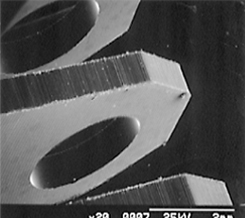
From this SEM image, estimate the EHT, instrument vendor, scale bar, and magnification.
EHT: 25 kV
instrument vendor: JEOL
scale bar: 2000000 nm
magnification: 0.02 K X